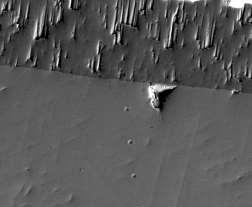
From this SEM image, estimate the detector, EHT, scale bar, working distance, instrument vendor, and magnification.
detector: ETD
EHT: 20 kV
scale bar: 50000 nm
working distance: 10 mm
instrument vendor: FEI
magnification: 1 K X